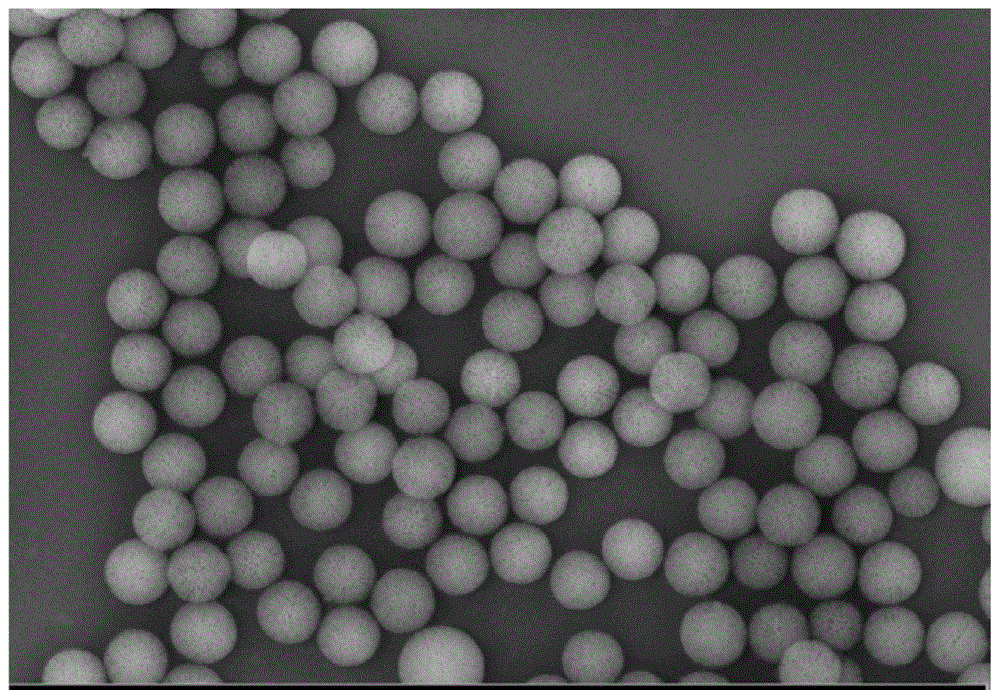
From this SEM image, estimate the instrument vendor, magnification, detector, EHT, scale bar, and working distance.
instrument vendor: Zeiss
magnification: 43.51 K X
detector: InLens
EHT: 5 kV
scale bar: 100 nm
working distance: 3.4 mm